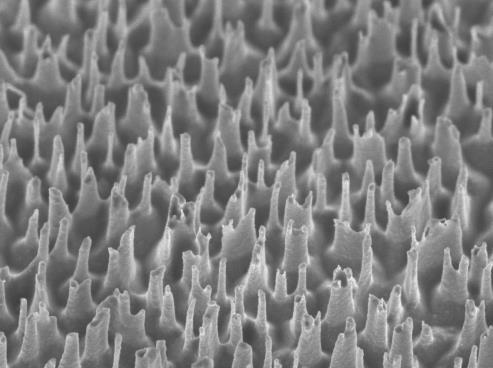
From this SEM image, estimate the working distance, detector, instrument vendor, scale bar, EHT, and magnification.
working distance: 3.2 mm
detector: SEI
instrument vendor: JEOL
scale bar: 1000 nm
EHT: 2 kV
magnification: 19 K X